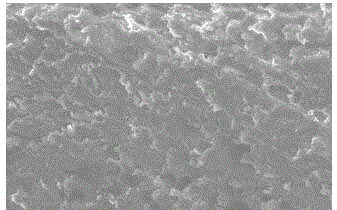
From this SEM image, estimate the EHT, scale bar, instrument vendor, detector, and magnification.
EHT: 10 kV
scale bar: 10000 nm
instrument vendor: JEOL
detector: SEI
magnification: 1 K X